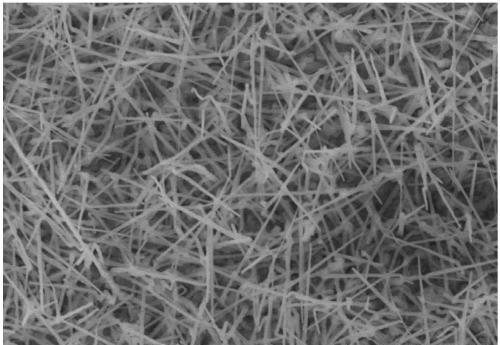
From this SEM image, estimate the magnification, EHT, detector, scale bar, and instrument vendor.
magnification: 20 K X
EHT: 10 kV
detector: SE(M)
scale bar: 2000 nm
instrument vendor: Hitachi